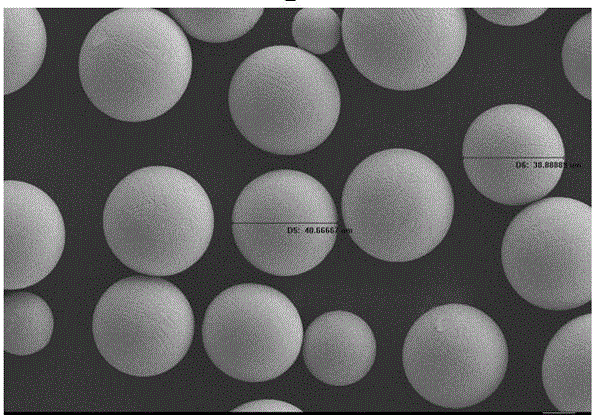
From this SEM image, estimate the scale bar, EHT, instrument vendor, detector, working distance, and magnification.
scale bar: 20000 nm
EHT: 15 kV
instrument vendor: Zeiss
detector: SE2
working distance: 7 mm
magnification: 0.5 K X